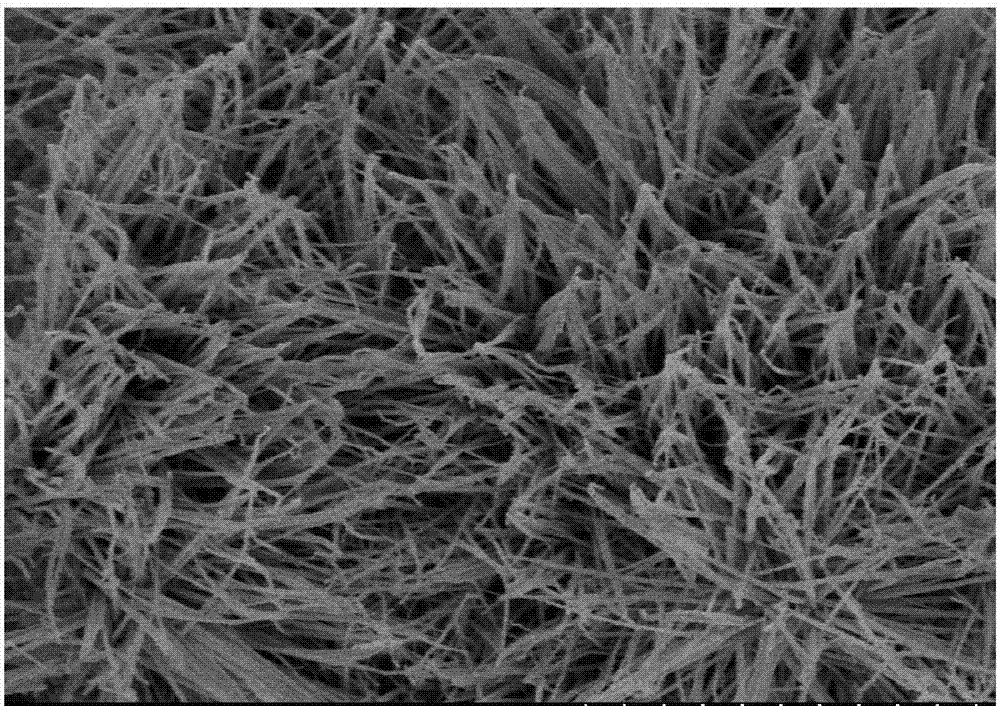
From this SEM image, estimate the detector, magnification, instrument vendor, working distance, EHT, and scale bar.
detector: SE(U)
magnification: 10 K X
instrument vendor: Hitachi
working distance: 8.9 mm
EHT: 3 kV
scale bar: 5000 nm